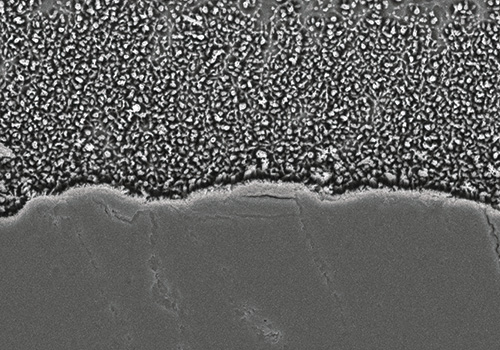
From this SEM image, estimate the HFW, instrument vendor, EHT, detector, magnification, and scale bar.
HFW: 12 µm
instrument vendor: Hitachi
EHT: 5 kV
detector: A+B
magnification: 10 K X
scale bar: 2000 nm